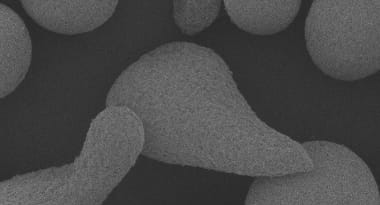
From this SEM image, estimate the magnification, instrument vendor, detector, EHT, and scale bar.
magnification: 0.4 K X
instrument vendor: JEOL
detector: SED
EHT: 5 kV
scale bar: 50000 nm